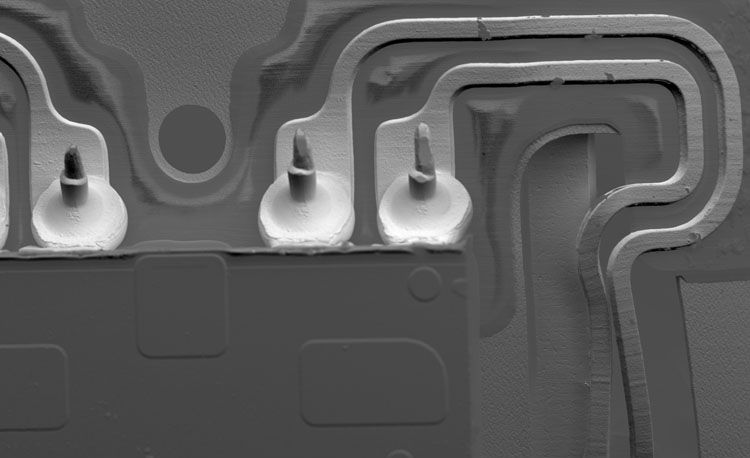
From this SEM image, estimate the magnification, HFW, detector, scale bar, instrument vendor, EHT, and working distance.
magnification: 0.39 K X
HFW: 1320 µm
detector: BSD Topo A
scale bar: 300000 nm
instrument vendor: Thermo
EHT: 10 kV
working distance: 6.141 mm